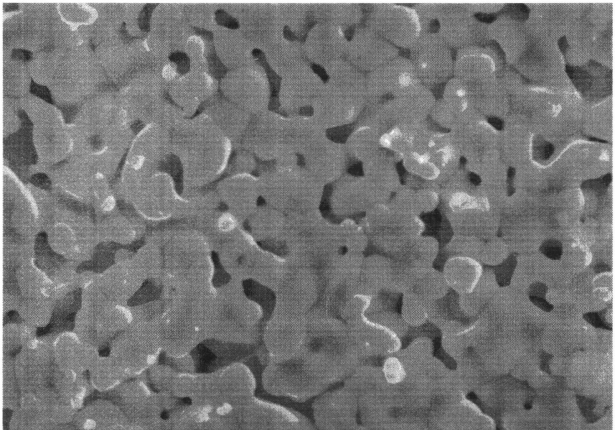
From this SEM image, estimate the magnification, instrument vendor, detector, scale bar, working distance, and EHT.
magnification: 10 K X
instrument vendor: Hitachi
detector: SE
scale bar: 5000 nm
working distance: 9.7 mm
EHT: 15 kV